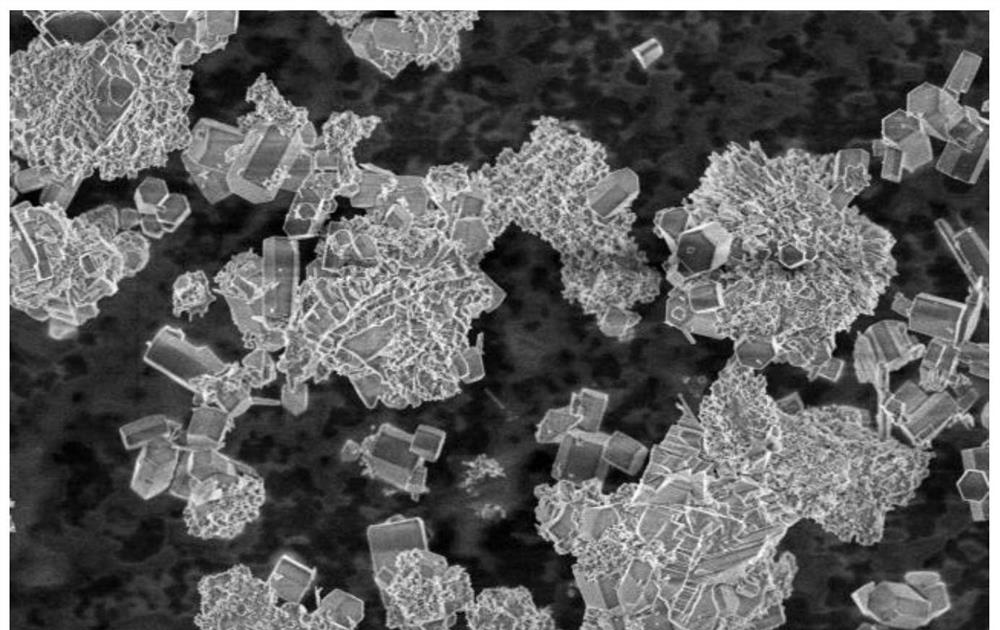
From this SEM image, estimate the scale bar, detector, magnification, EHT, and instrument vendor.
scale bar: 20000 nm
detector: SE(M)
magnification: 2 K X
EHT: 3 kV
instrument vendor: Hitachi